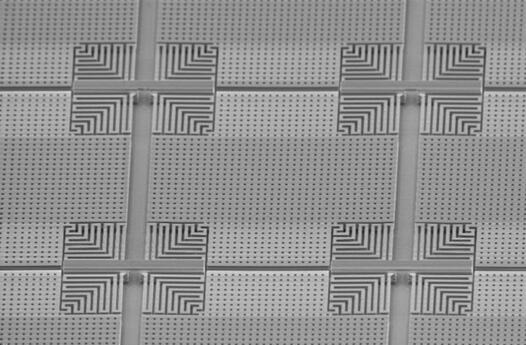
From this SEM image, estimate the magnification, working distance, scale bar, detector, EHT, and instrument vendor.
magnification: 1.2 K X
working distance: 13 mm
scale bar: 20000 nm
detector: SE2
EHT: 3 kV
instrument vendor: Zeiss